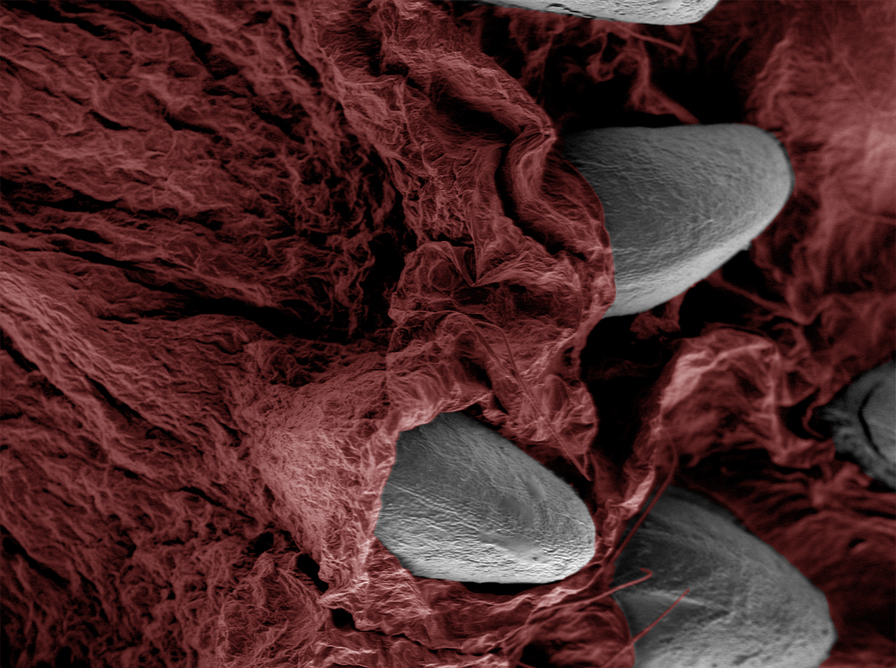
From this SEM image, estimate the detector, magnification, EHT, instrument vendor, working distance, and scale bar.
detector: LVSED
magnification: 0.035 K X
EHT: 15 kV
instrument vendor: JEOL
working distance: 15.8 mm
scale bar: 500000 nm